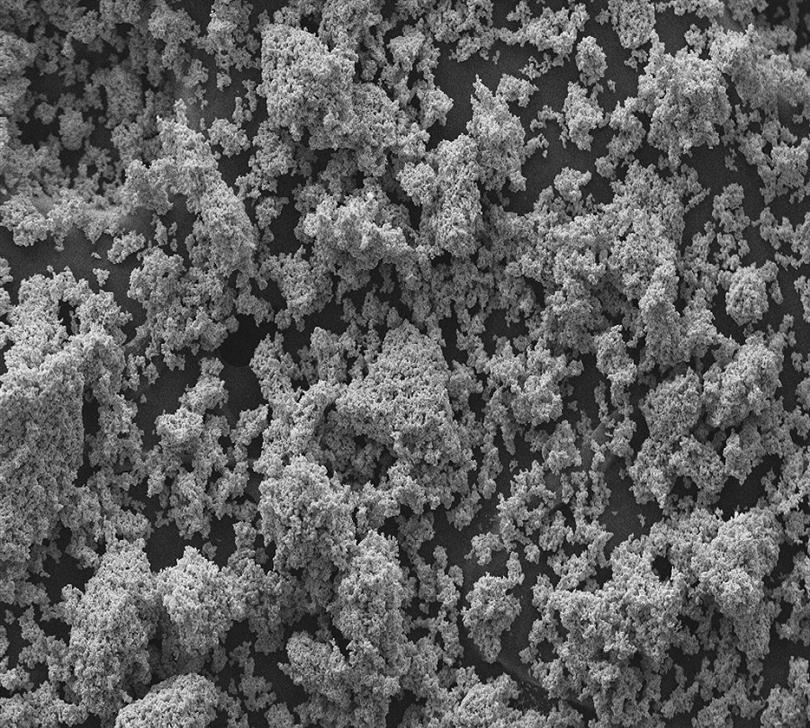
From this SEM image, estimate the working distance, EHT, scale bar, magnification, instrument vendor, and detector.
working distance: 8 mm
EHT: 20 kV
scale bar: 20000 nm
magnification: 0.5 K X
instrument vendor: Zeiss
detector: SE1